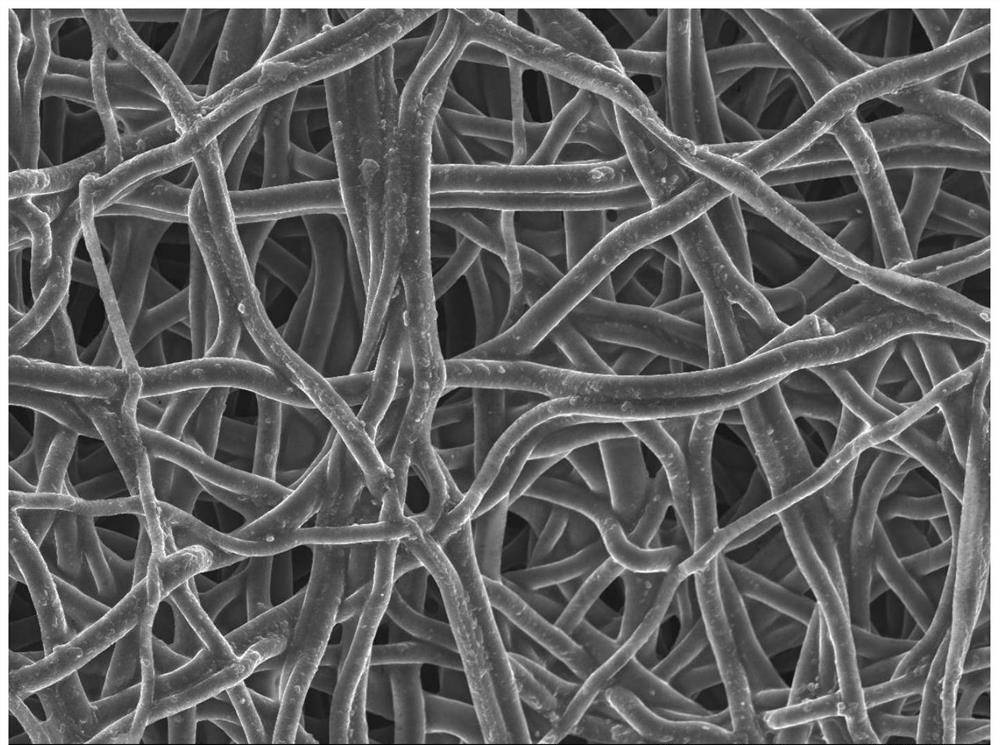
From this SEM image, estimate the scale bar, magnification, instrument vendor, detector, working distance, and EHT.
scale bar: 1000 nm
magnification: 3 K X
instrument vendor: JEOL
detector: SEI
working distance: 7.9 mm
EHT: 5 kV